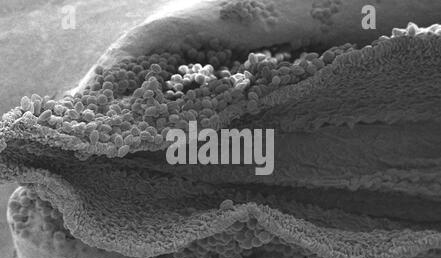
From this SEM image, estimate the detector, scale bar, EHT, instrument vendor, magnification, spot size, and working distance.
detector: SE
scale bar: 200000 nm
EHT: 12 kV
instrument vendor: FEI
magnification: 0.103 K X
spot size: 3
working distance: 7.1 mm